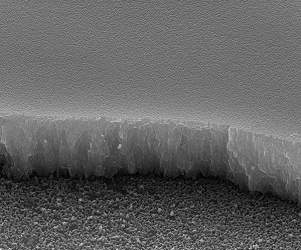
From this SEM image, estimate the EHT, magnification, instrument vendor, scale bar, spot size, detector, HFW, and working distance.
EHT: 30 kV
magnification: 30 K X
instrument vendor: FEI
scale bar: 4000 nm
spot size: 1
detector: ETD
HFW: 9.95 µm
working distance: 10 mm